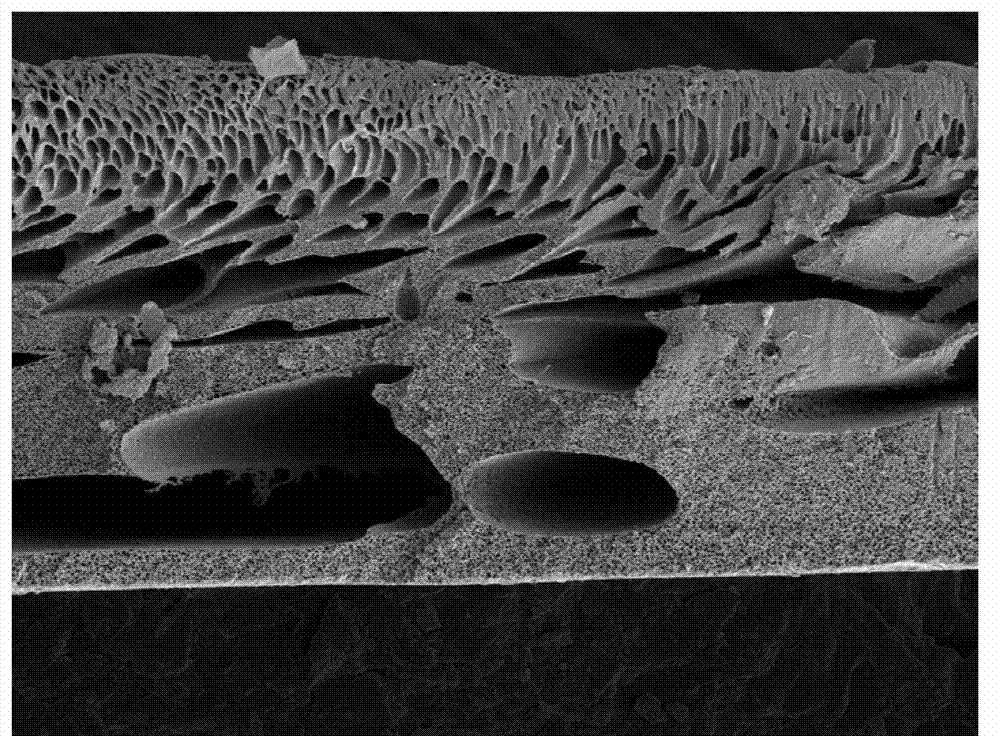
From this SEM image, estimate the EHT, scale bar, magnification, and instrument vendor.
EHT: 5 kV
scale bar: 10000 nm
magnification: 0.5 K X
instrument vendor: Hitachi